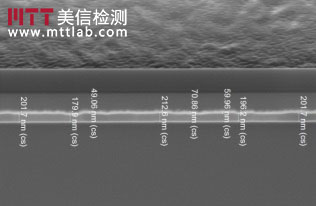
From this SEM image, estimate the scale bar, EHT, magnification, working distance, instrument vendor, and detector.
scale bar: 3000 nm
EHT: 1 kV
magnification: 35 K X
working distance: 3 mm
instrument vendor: FEI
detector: SE